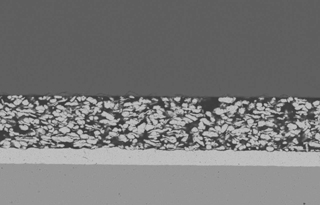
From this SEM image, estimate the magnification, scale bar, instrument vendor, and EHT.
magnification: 1 K X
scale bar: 10000 nm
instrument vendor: JEOL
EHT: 15 kV